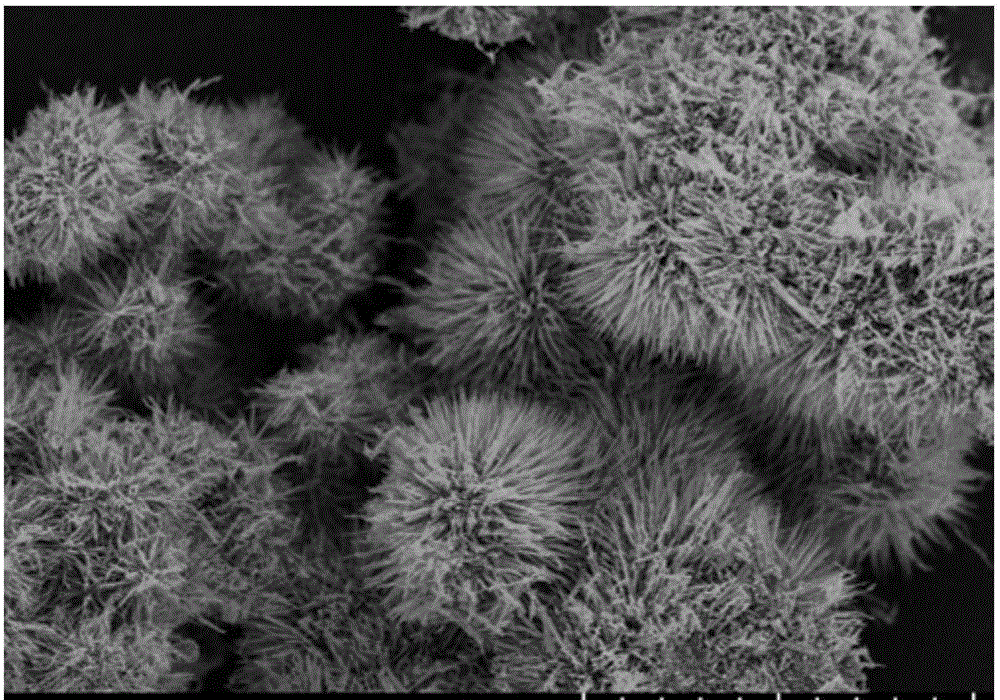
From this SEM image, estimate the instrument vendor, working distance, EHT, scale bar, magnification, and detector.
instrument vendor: Hitachi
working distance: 8.3 mm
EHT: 3 kV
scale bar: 5000 nm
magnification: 10 K X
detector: SE(UL)